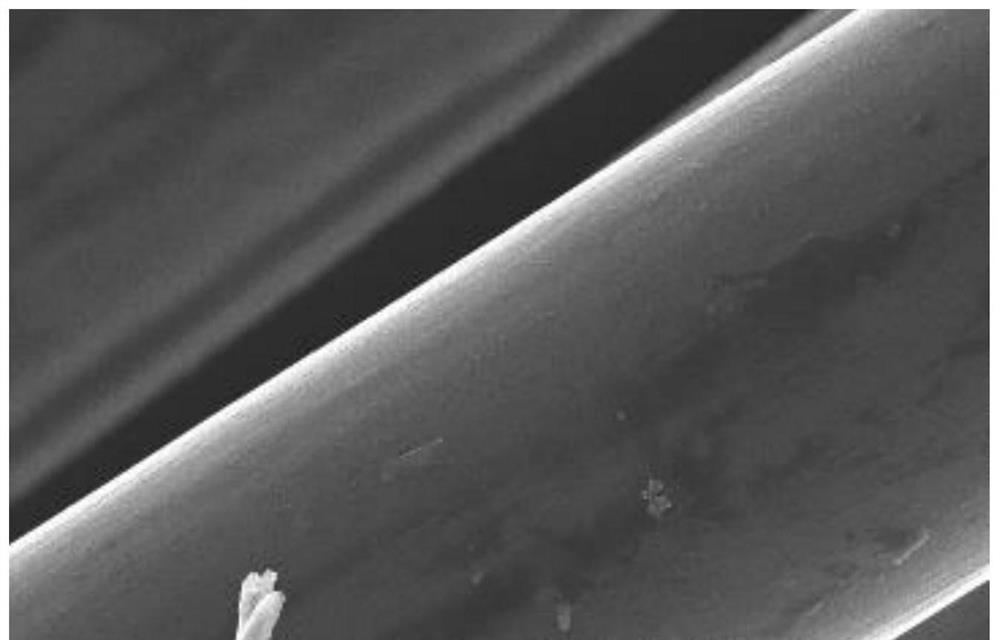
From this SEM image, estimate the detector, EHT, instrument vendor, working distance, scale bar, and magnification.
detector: SE(M)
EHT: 5 kV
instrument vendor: Hitachi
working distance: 7.6 mm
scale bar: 5000 nm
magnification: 10 K X